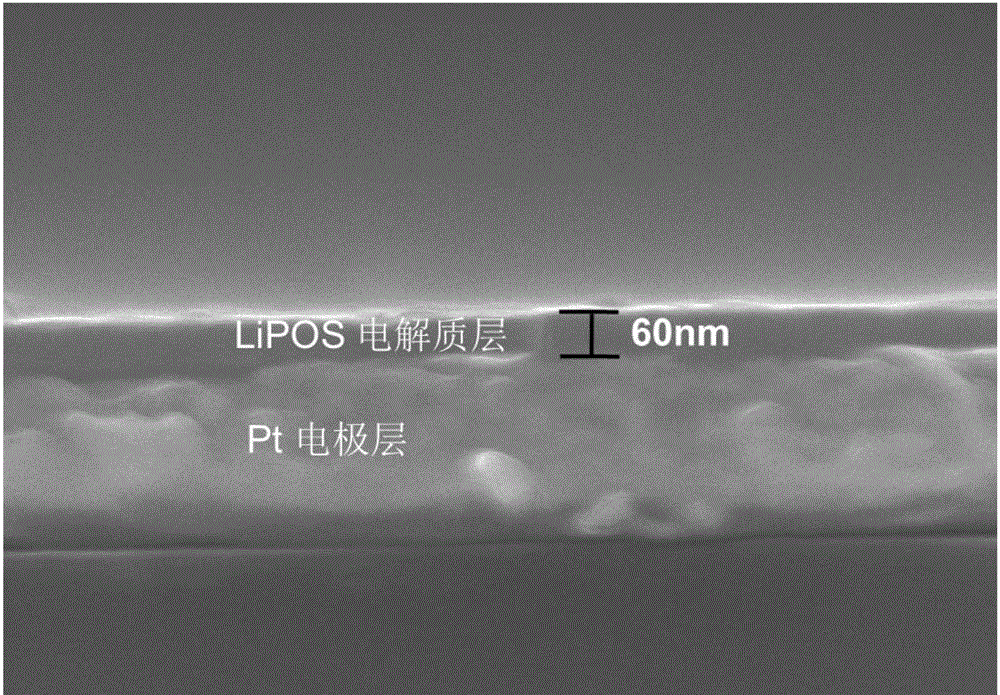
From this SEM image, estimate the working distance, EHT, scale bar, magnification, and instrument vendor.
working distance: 5.9 mm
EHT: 10 kV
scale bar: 500 nm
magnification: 100 K X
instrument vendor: Hitachi